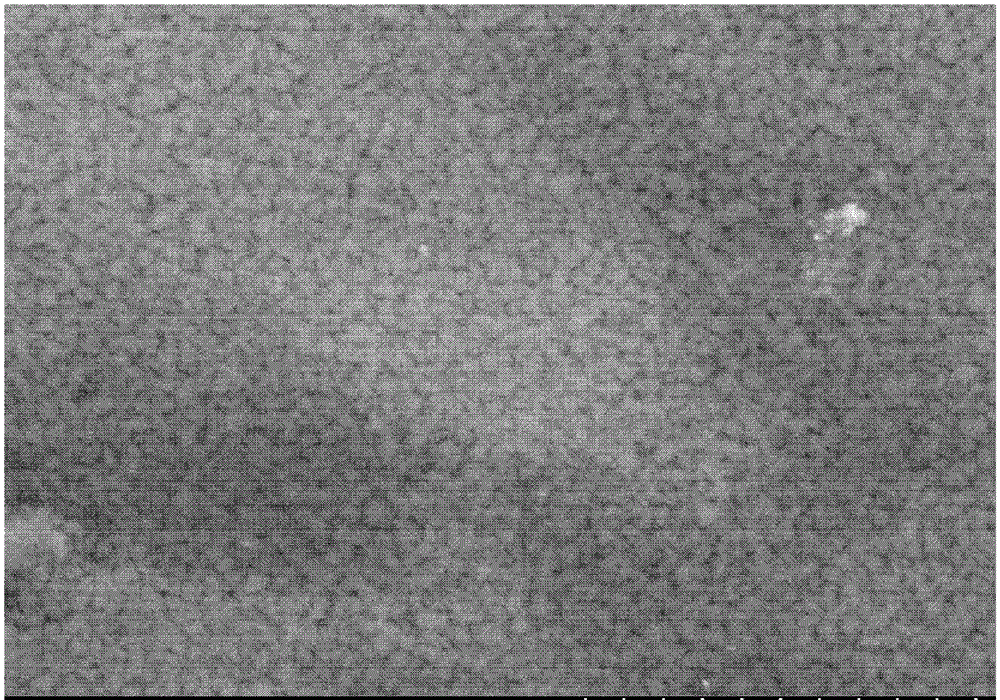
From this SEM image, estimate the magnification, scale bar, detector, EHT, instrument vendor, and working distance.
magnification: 10 K X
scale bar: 5000 nm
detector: SE(M,LA0)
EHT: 15 kV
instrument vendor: Hitachi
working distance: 15.1 mm